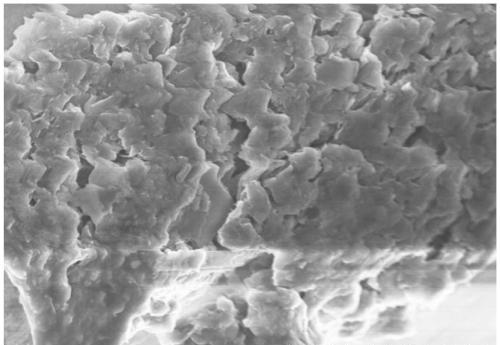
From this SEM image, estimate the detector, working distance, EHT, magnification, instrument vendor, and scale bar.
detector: SE(M)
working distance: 8.1 mm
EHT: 5 kV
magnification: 10 K X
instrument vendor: Hitachi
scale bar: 5000 nm